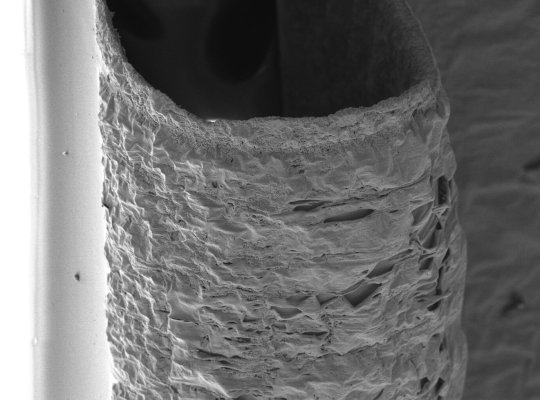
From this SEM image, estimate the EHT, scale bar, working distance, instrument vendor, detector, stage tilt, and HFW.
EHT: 10 kV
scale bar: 300000 nm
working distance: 10 mm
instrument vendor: FEI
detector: ETD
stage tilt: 30°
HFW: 800 µm